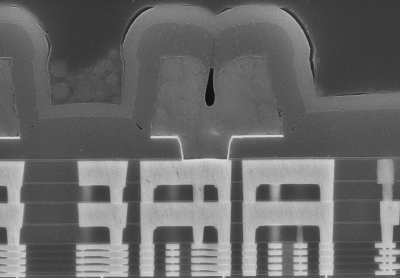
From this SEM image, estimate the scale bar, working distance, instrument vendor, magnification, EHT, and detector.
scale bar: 5000 nm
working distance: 8 mm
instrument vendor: Hitachi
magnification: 9 K X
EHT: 15 kV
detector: LA5(UL)